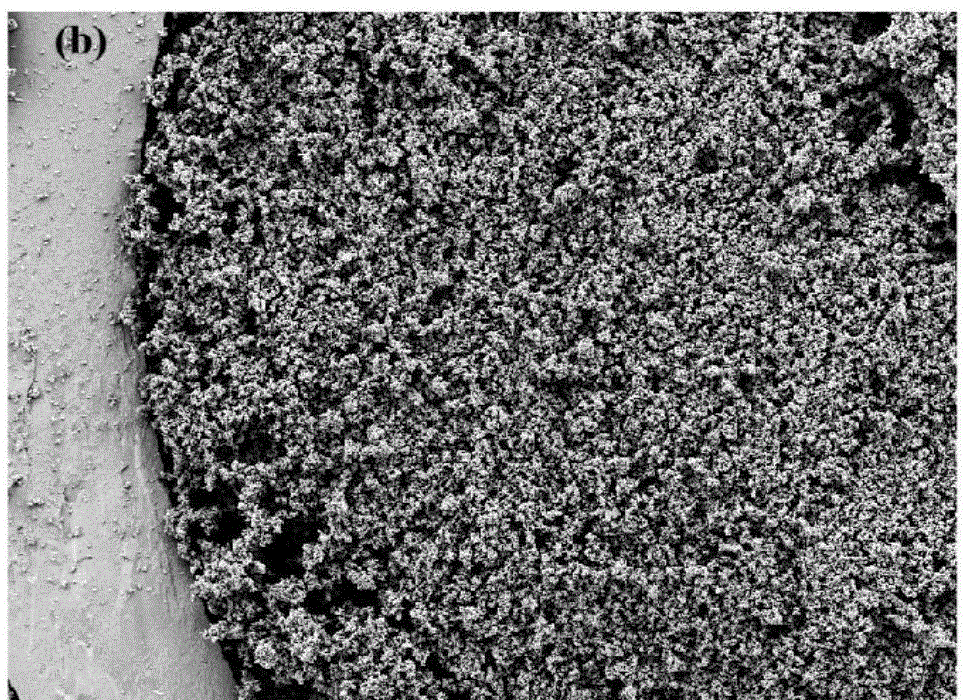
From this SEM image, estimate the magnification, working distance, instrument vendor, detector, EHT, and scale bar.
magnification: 1.15 K X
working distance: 7.3 mm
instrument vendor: Zeiss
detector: SE2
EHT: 5 kV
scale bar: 10000 nm